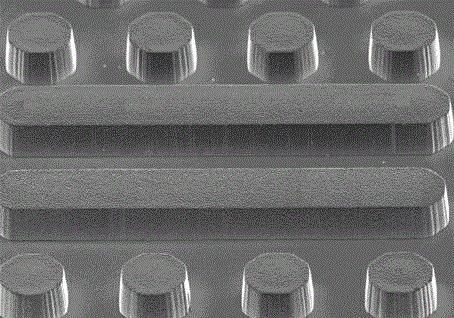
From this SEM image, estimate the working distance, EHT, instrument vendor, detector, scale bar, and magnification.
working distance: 24.5 mm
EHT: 15 kV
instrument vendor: Hitachi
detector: SE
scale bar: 200000 nm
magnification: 0.2 K X